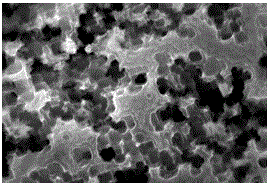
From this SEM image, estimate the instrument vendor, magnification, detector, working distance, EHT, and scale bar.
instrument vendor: Zeiss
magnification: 50 K X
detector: InLens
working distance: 7.6 mm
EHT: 5 kV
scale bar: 100 nm